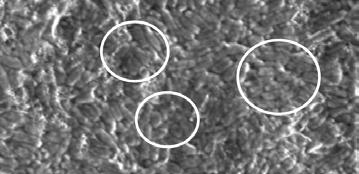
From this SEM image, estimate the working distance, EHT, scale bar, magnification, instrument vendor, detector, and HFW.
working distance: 9.7 mm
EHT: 5 kV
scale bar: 10000 nm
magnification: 10 K X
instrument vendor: FEI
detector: LFD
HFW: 29.8 µm